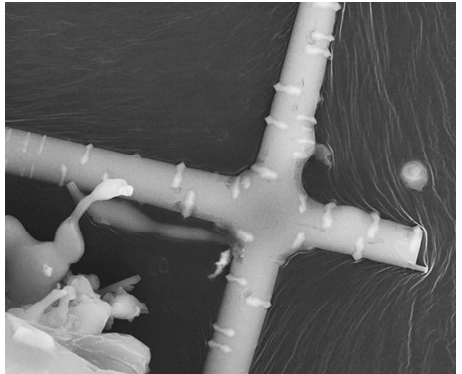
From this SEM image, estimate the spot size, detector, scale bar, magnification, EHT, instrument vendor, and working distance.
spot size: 5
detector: None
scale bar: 2000 nm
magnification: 20 K X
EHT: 20 kV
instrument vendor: FEI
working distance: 5.5 mm